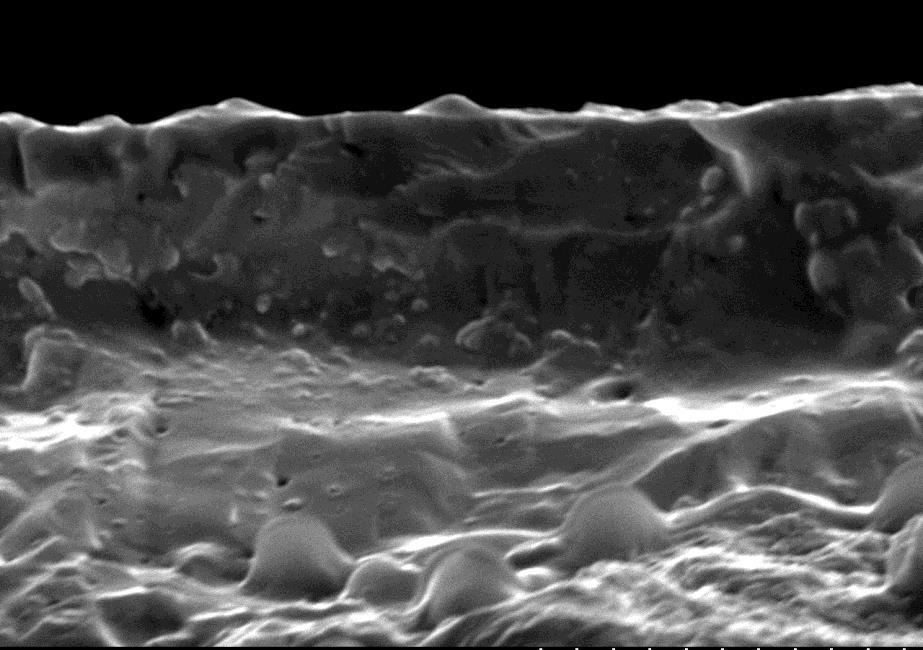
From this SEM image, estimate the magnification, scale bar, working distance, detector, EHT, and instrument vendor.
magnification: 5 K X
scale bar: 10000 nm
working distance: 10.4 mm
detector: SE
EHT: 15 kV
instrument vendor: Hitachi